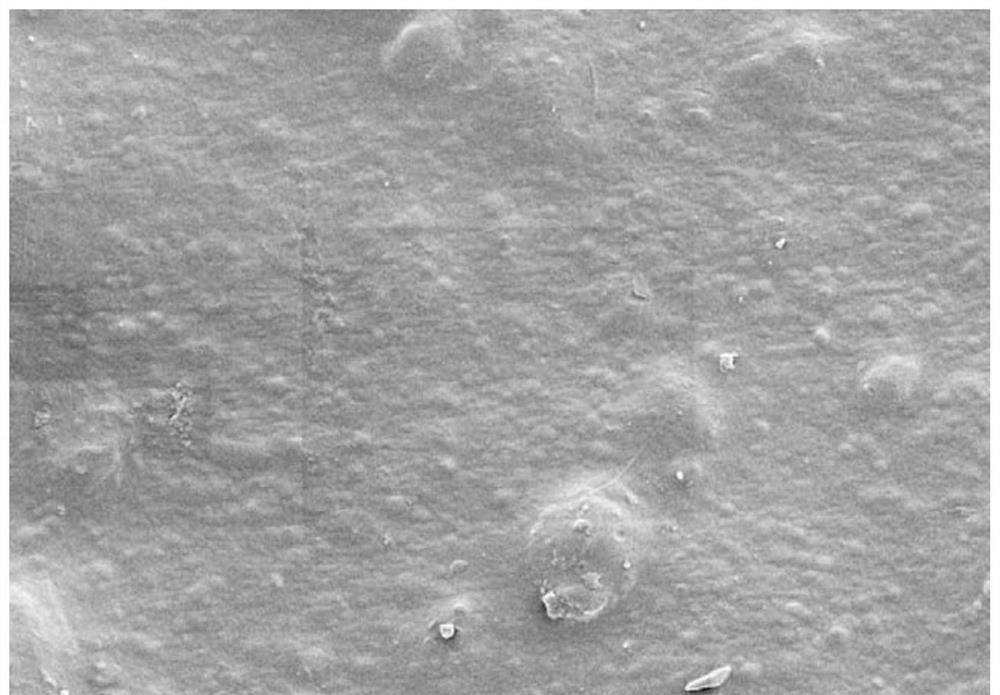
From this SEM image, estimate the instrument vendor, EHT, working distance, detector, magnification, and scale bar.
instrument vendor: Zeiss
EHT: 3 kV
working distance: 9.2 mm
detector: SE2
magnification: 2 K X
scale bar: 10000 nm